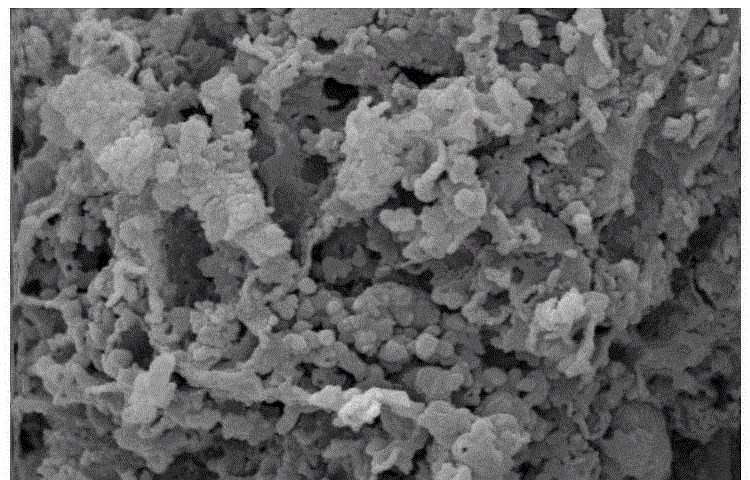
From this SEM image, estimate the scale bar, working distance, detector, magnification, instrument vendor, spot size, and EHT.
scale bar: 1000 nm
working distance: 5.3 mm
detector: SE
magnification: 20 K X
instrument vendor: FEI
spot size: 3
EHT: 20 kV